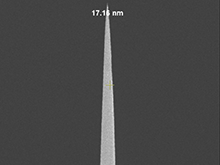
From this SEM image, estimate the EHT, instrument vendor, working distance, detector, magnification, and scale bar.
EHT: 3 kV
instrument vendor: FEI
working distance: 5.1 mm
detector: TLD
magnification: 50 K X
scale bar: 2000 nm